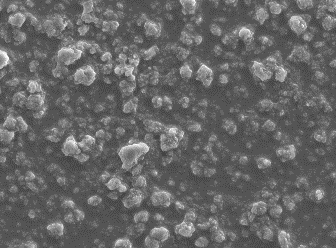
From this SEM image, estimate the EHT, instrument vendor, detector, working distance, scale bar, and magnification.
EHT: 15 kV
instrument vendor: JEOL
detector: SEC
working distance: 10 mm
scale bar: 10000 nm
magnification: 1 K X